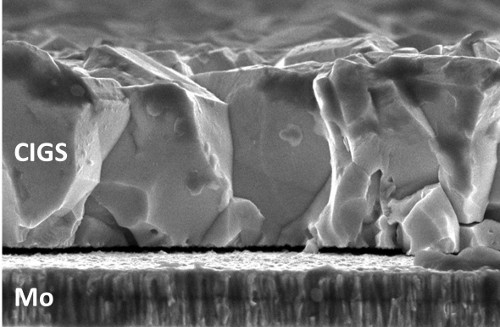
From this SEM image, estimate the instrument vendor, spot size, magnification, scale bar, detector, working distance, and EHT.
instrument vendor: FEI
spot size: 3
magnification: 50 K X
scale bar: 1000 nm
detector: TLD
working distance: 5 mm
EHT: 5 kV